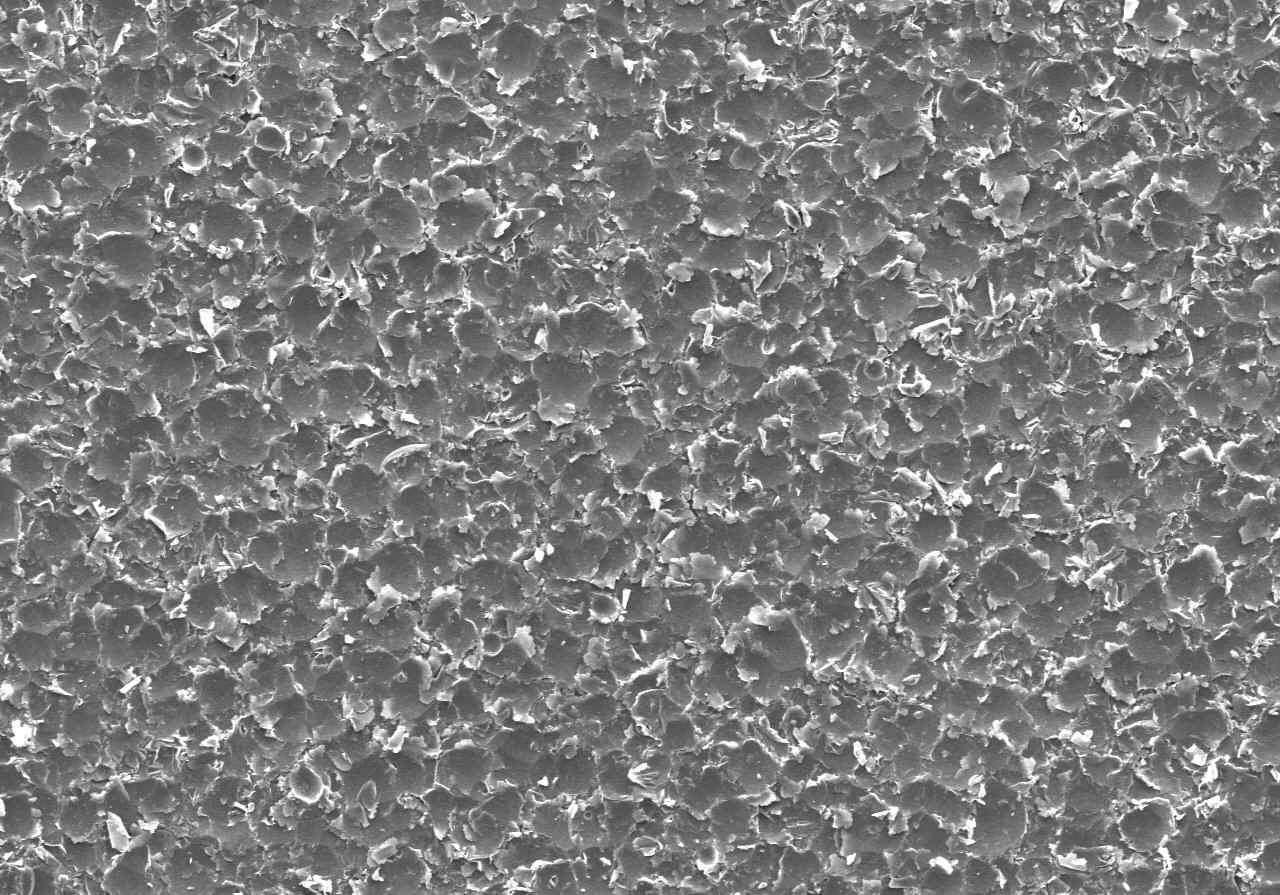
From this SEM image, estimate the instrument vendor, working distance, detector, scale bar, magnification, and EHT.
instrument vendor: Hitachi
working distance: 9.7 mm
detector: SE(U)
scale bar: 500000 nm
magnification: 0.1 K X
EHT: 15 kV